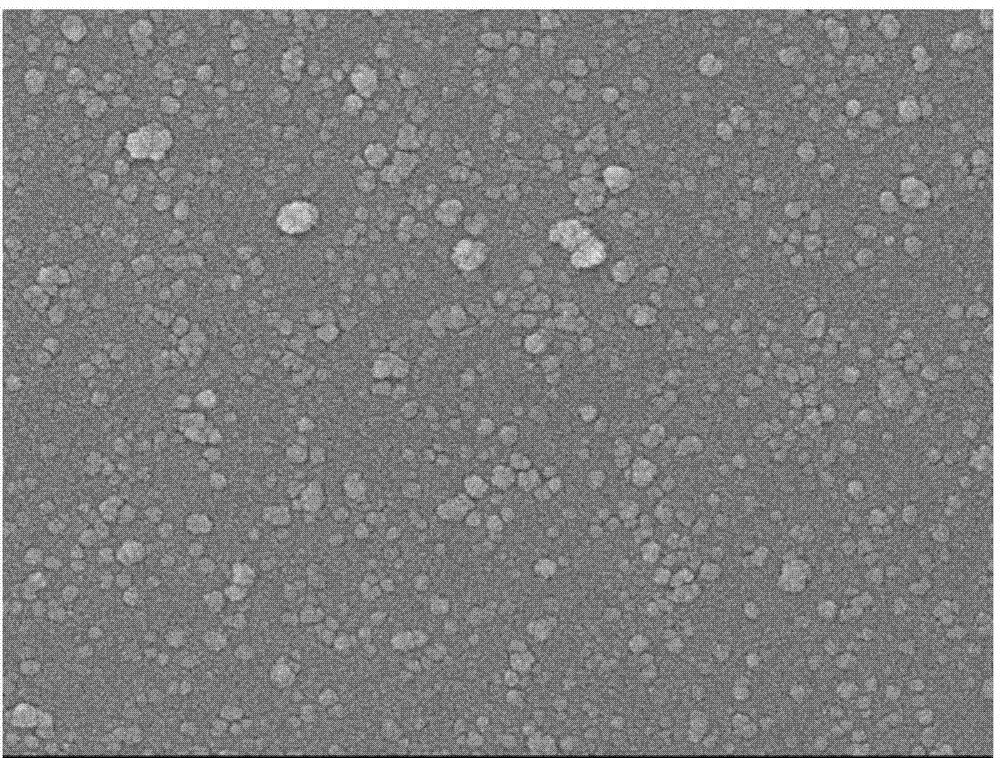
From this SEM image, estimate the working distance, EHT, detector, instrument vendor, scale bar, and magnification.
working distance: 7.6 mm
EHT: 10 kV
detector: SEI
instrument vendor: JEOL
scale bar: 100 nm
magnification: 30 K X